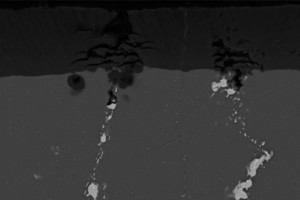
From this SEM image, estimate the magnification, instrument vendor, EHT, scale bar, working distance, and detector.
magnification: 0.75 K X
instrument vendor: Zeiss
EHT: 20 kV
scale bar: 20000 nm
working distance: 24 mm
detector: BSD